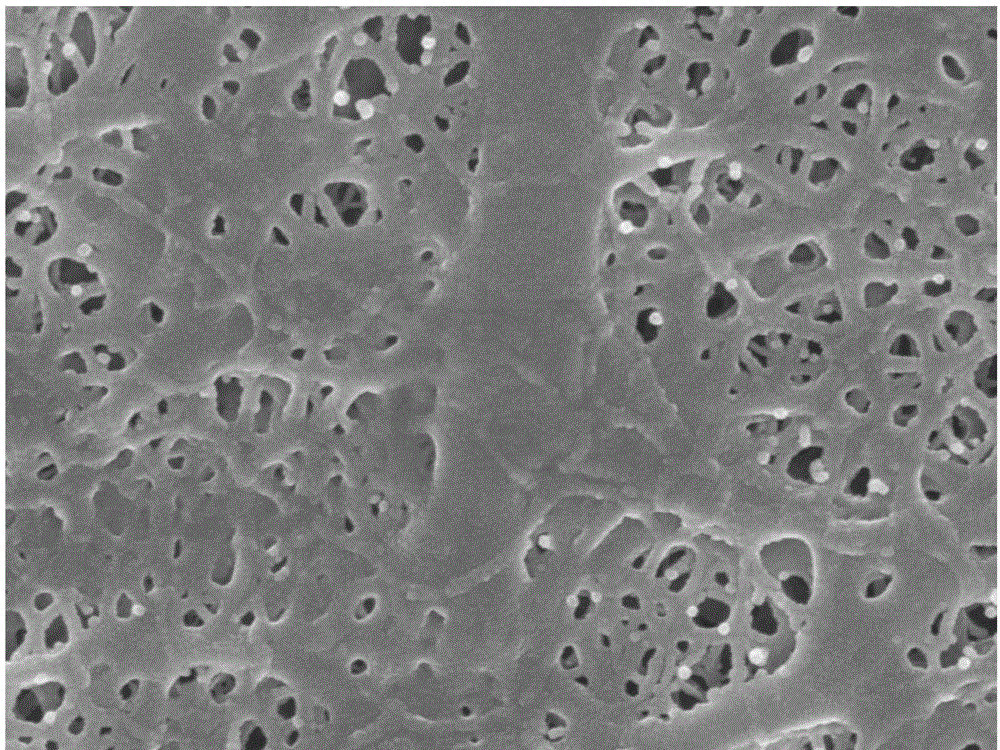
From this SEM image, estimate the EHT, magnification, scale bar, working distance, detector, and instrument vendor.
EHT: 10 kV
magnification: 50 K X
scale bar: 100 nm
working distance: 9.6 mm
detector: SEI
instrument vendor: JEOL